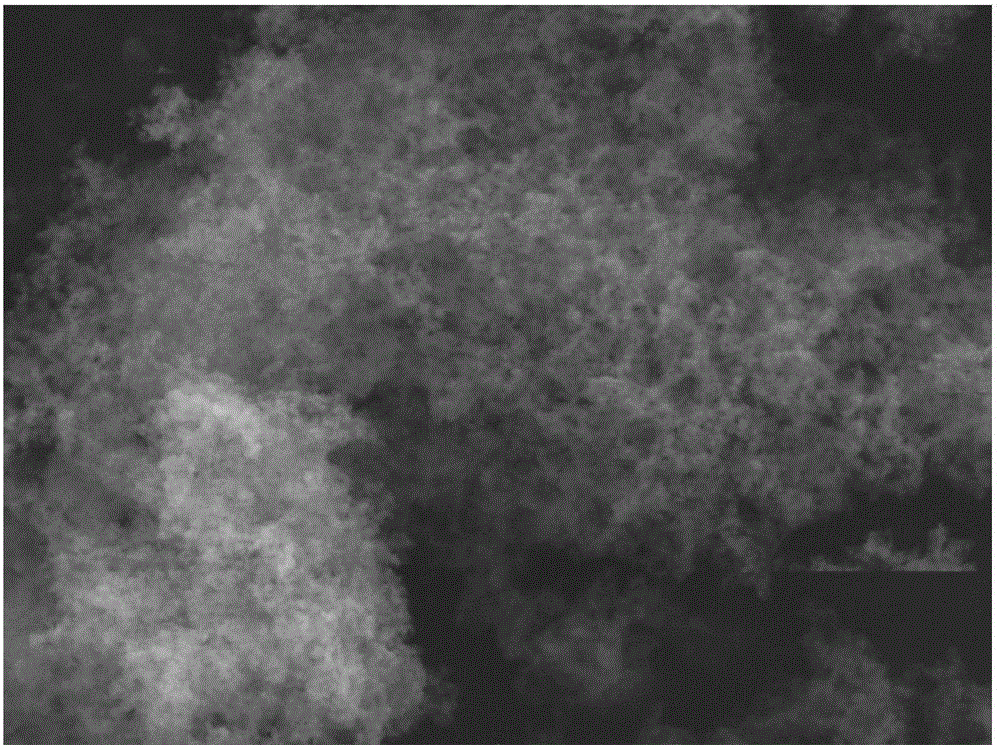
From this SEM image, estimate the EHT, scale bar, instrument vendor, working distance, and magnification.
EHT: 20 kV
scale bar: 5000 nm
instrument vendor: Tescan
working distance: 14.48 mm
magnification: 10 K X